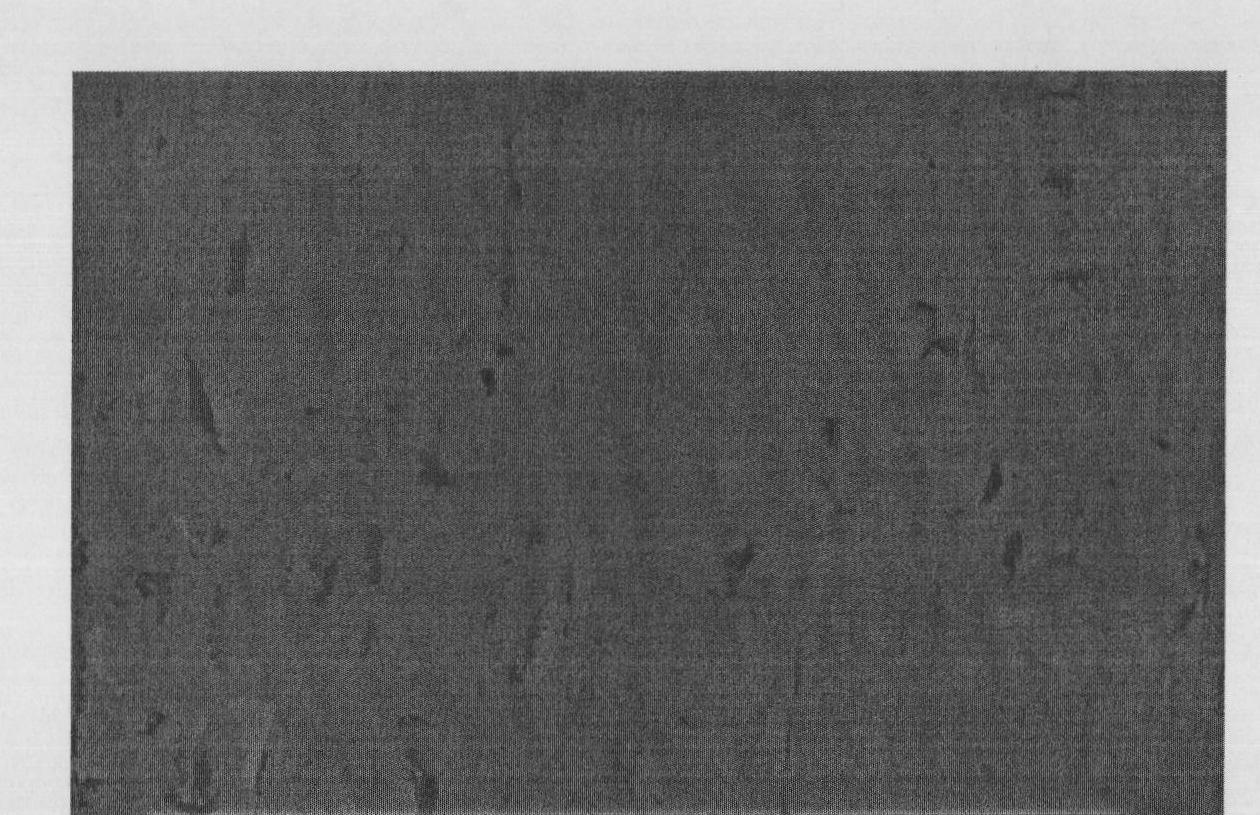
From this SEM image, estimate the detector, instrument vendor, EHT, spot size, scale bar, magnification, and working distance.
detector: SE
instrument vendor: FEI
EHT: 20 kV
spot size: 3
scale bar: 20000 nm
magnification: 1 K X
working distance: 10 mm